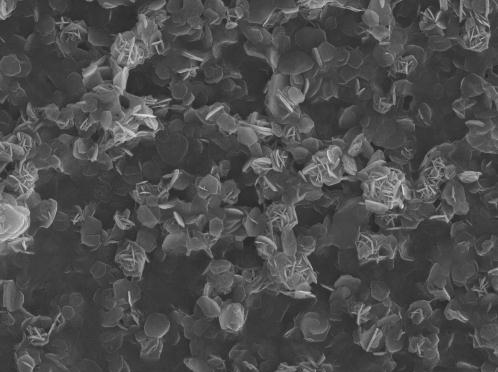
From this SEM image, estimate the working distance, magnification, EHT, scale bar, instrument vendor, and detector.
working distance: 15.2 mm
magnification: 3 K X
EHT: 20 kV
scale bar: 1000 nm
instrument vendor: JEOL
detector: SEI